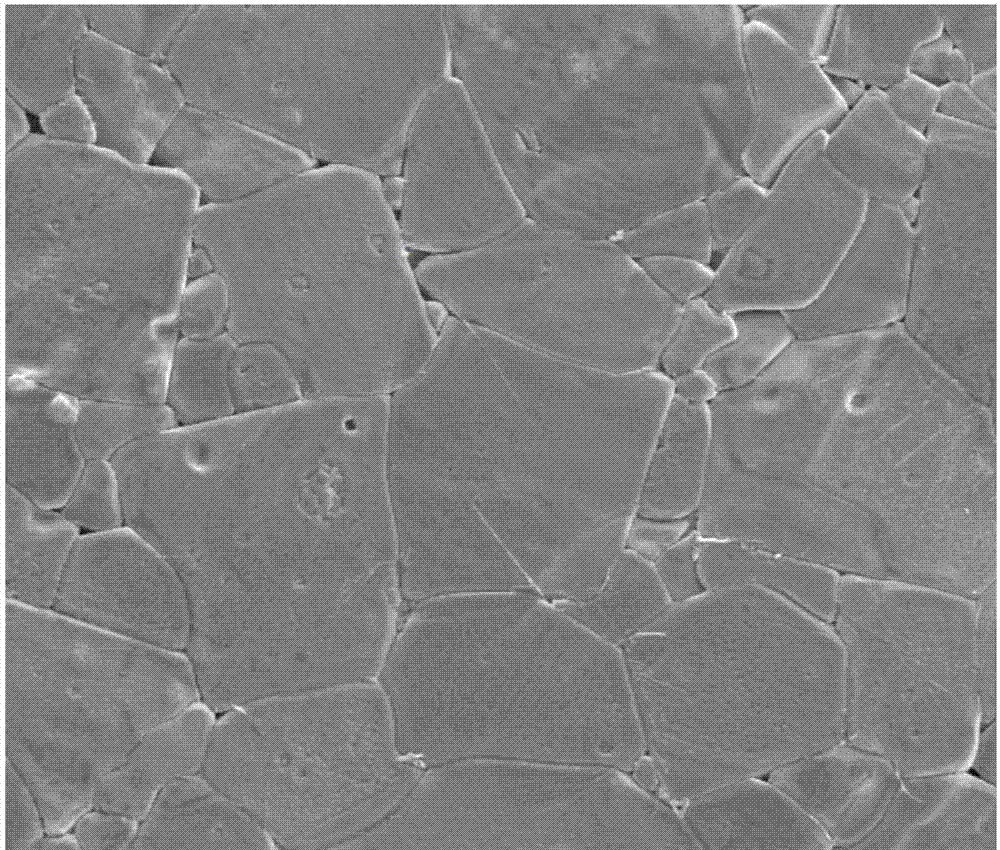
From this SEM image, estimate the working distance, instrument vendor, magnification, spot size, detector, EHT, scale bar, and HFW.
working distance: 11.1 mm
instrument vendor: FEI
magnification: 1 K X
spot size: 5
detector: SE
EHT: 20 kV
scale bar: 100000 nm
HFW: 270 µm